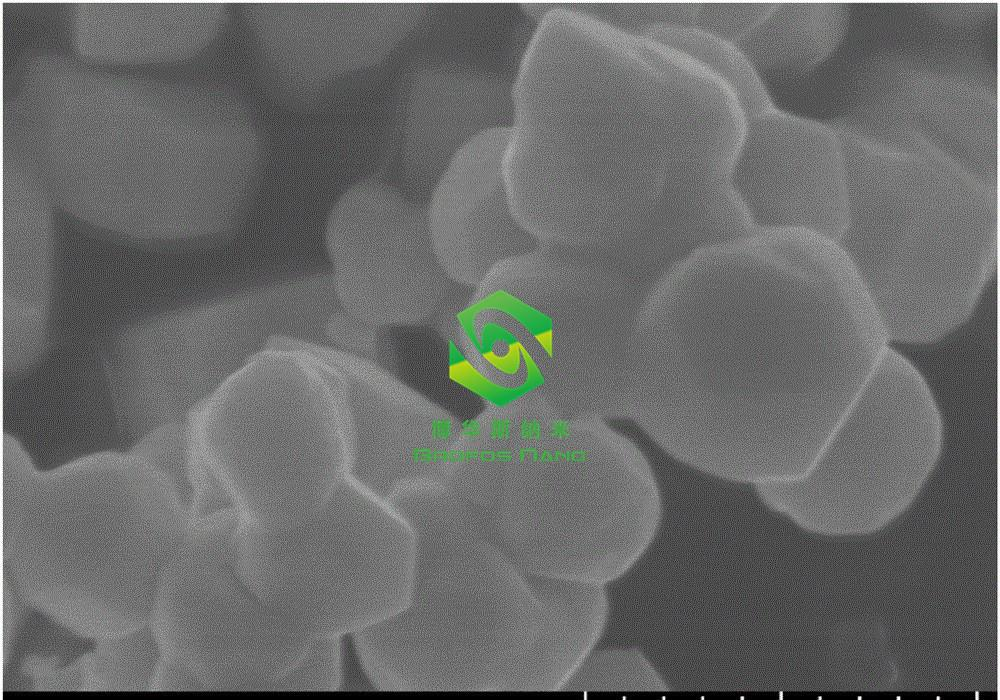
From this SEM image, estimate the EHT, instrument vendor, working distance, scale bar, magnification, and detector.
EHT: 10 kV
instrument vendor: Hitachi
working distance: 10.6 mm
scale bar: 500 nm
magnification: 100 K X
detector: SE(U)